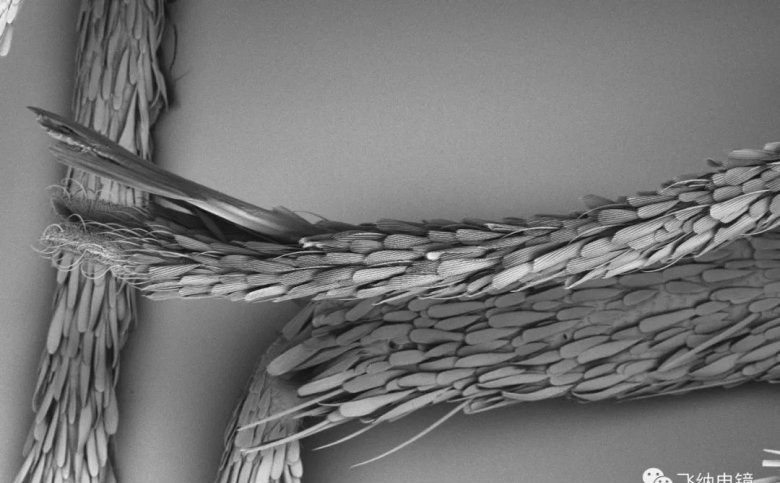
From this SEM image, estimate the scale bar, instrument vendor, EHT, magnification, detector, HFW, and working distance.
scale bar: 200000 nm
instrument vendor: other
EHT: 5 kV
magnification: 0.17 K X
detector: BSD Full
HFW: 753 µm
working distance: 7.8 mm